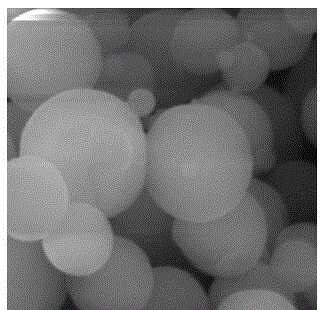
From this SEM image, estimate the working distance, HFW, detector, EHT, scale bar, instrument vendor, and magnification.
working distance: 11.66 mm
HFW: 10.4 µm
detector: SE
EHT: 20 kV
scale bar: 2000 nm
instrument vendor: Tescan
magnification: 20 K X